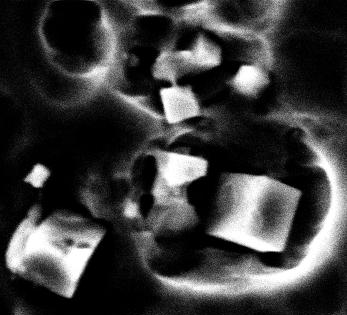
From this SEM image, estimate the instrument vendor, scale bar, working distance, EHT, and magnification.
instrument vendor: Tescan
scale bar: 6000 nm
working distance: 26.1 mm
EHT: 30 kV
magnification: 3.722 K X